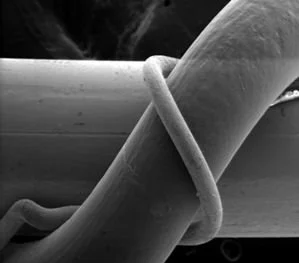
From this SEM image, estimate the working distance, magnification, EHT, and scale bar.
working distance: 20 mm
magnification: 0.06 K X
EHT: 20 kV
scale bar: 100000 nm